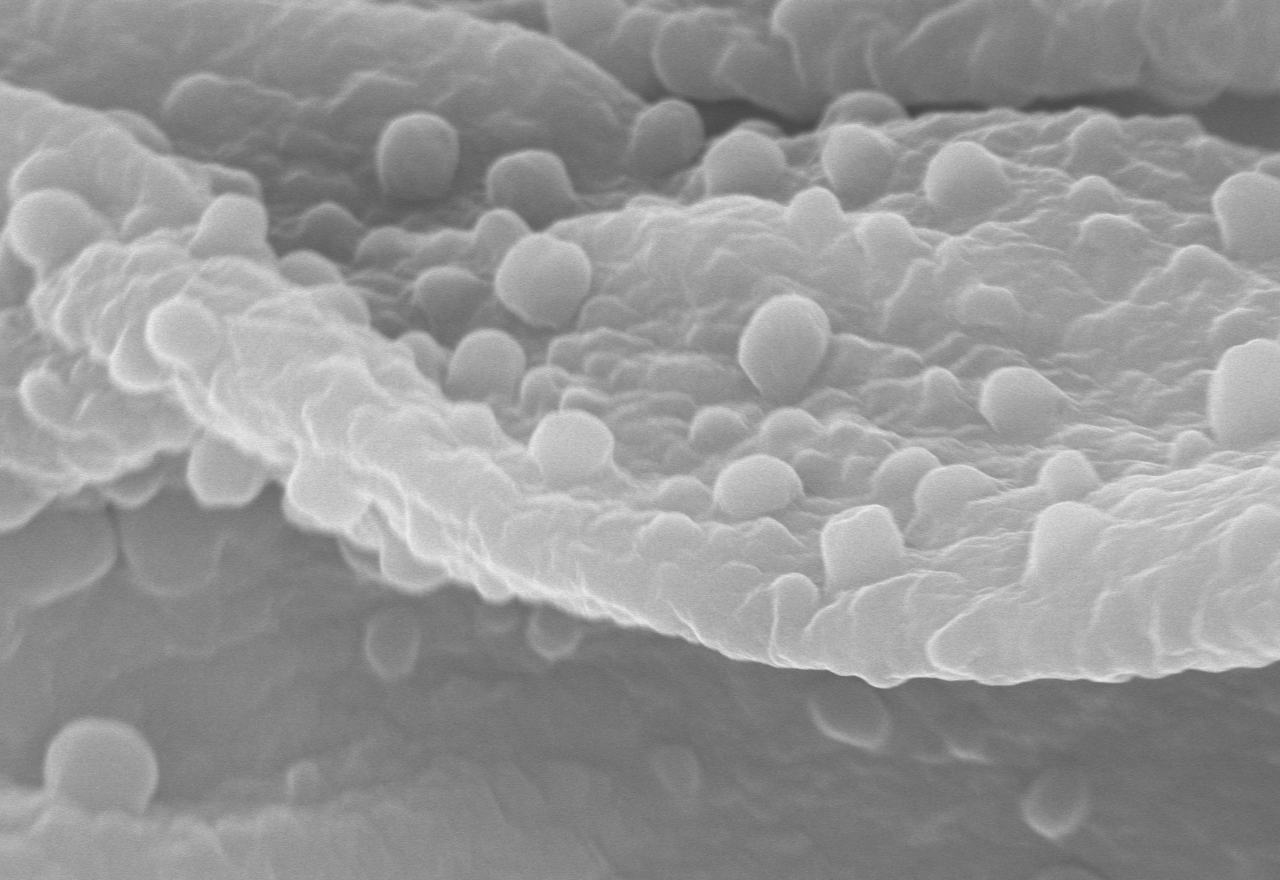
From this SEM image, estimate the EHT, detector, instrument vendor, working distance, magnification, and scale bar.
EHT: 20 kV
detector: SE(U)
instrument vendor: Hitachi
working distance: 8.7 mm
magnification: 20 K X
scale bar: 2000 nm